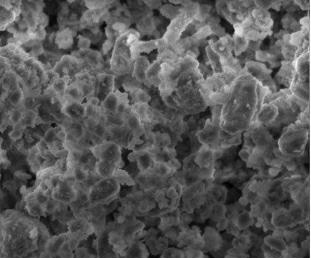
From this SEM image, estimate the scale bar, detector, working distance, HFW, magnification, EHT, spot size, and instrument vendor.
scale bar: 20000 nm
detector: ETD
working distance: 8.9 mm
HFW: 67.6 µm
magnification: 2 K X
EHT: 15 kV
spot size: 4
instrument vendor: FEI